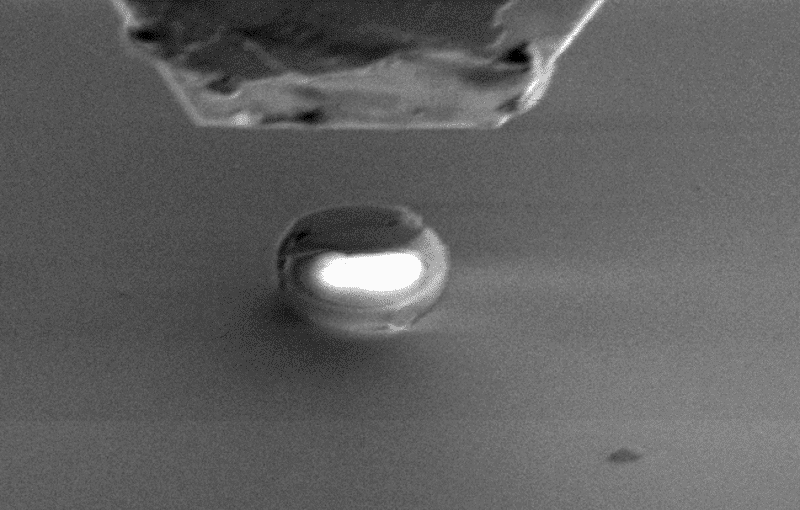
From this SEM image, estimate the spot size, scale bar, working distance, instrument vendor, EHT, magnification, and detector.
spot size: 3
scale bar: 5000 nm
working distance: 10 mm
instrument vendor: FEI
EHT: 3 kV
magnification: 5 K X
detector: SE